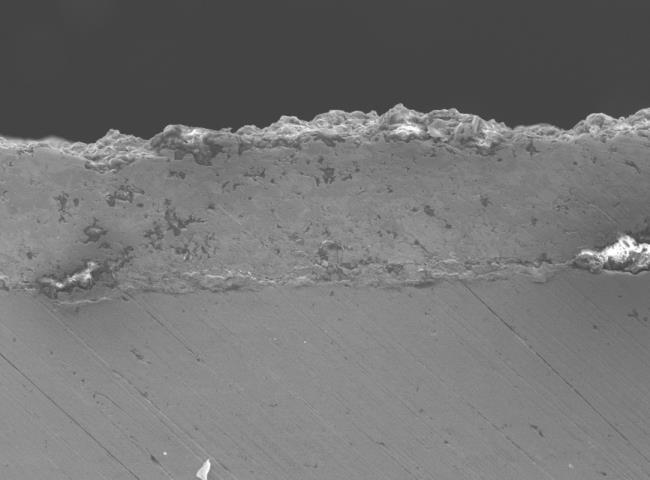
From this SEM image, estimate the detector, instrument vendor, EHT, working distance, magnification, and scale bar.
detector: SEI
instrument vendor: JEOL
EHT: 15 kV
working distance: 10 mm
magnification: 0.15 K X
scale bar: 100000 nm